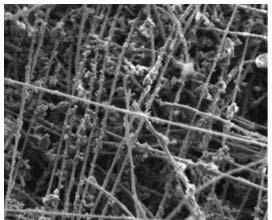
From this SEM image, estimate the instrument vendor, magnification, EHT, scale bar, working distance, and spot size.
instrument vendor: FEI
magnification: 3.352 K X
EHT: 5 kV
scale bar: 30000 nm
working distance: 11.1 mm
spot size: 5